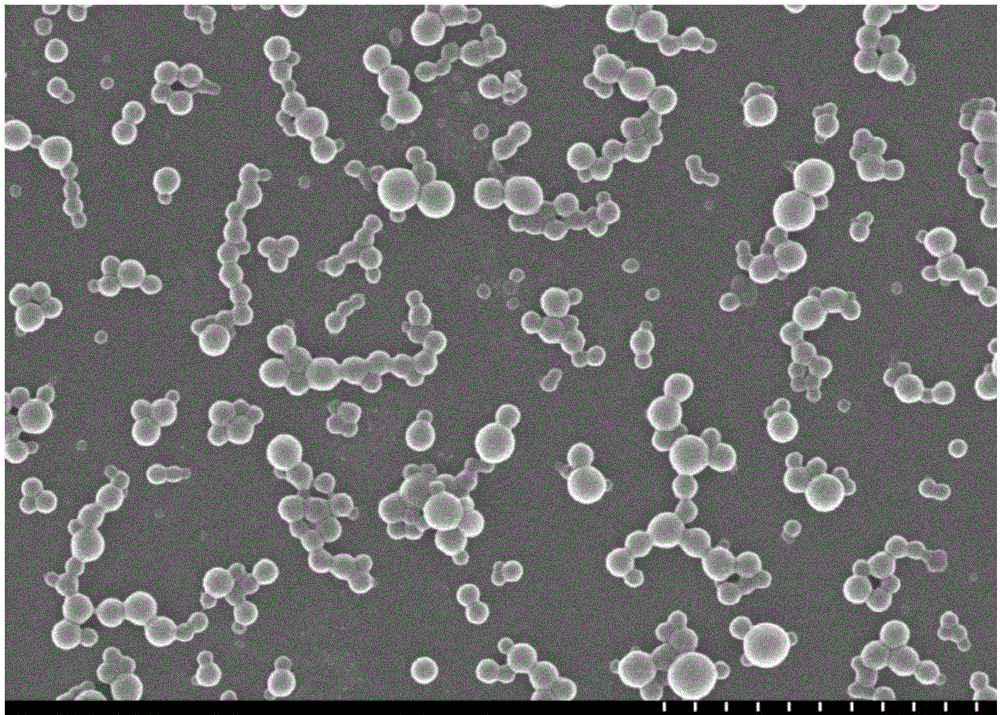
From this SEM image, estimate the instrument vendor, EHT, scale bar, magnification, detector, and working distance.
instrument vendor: Hitachi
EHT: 10 kV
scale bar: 1000 nm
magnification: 40 K X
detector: SE(U)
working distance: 14.1 mm